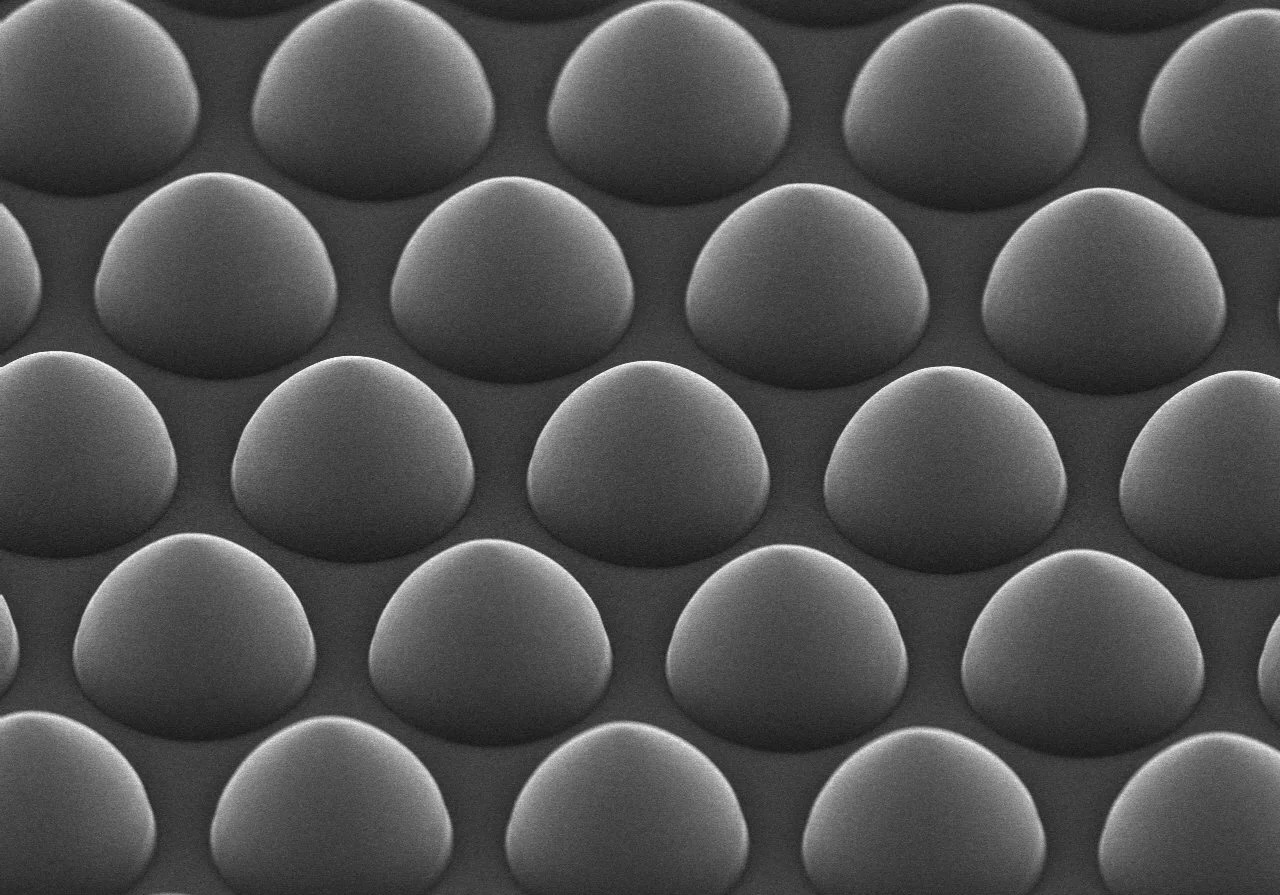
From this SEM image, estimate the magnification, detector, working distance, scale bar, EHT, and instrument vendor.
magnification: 10 K X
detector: SE(U)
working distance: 6.2 mm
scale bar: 5000 nm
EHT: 15 kV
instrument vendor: Hitachi